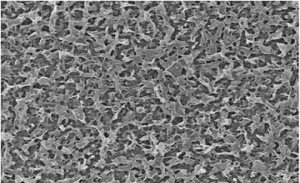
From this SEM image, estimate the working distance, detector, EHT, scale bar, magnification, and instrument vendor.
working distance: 3.7 mm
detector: SE(M,LA0)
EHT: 5 kV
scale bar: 5000 nm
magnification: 10 K X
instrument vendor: Hitachi